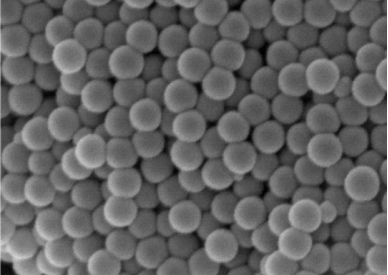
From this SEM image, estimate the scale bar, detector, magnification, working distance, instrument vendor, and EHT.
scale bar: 200 nm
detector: InLens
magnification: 50 K X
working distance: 6 mm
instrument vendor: Zeiss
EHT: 5 kV